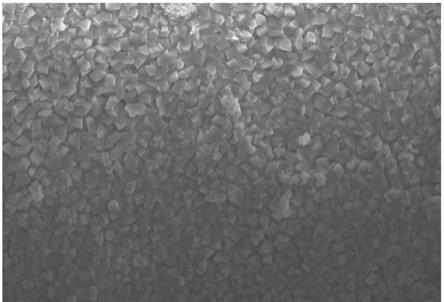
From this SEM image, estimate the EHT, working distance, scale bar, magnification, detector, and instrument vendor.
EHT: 20 kV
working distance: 8.8 mm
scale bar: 50000 nm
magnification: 1 K X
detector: SE(U)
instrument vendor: Hitachi